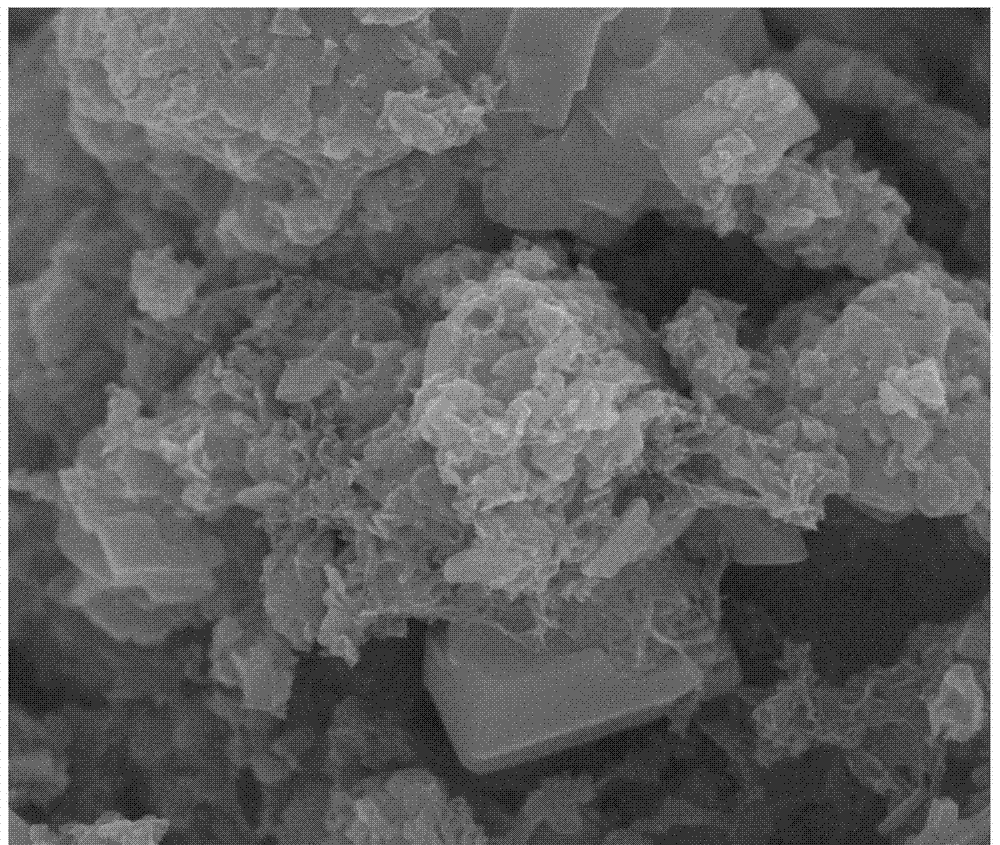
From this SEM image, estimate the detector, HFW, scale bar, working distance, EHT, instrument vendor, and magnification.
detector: TLD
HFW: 4.8 µm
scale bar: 1000 nm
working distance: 4.9 mm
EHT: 15 kV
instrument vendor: FEI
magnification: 50 K X